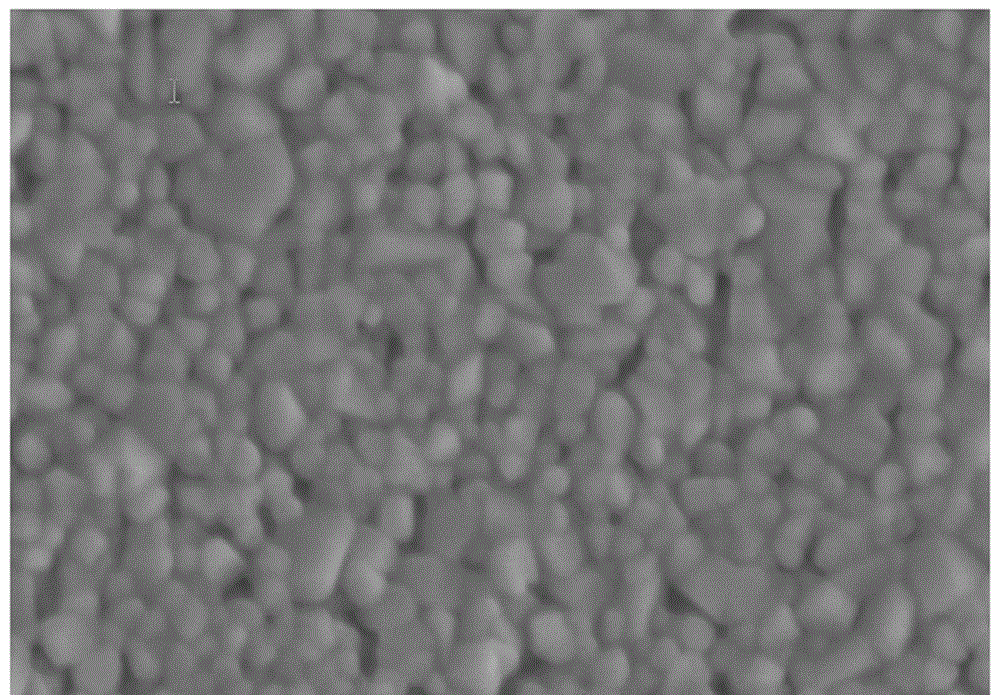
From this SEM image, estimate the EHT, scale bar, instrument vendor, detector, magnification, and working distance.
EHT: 3 kV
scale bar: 500 nm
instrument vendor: Hitachi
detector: SE(M)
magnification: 100 K X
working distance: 2.8 mm